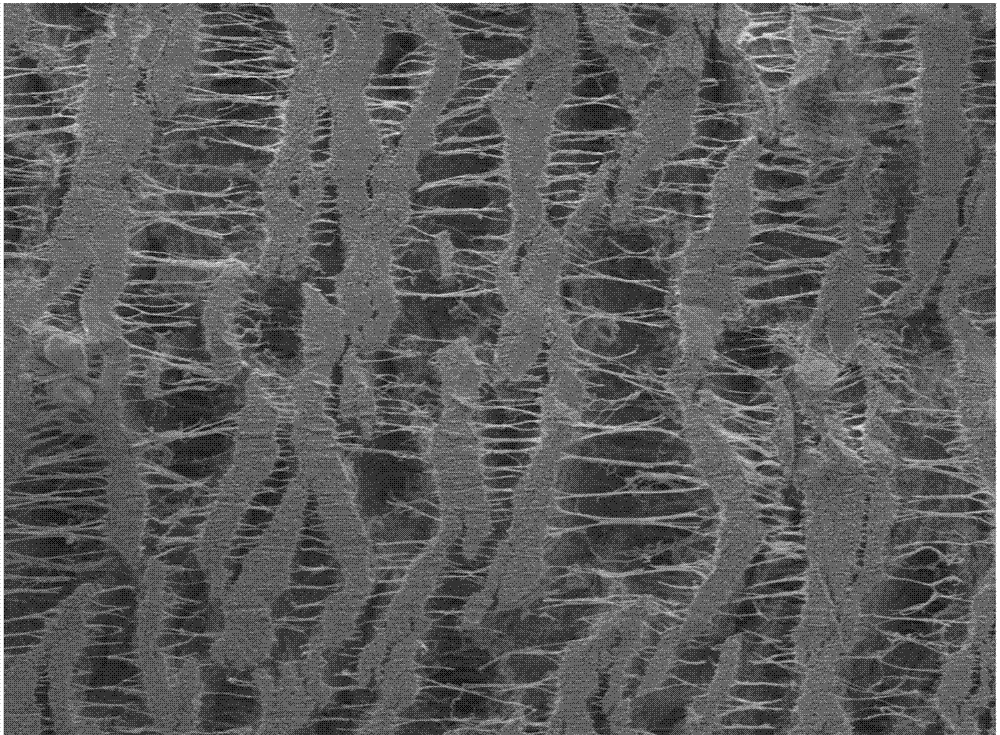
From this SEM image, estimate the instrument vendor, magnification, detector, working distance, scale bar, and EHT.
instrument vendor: JEOL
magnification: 0.5 K X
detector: LED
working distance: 8.1 mm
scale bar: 10000 nm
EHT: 3 kV